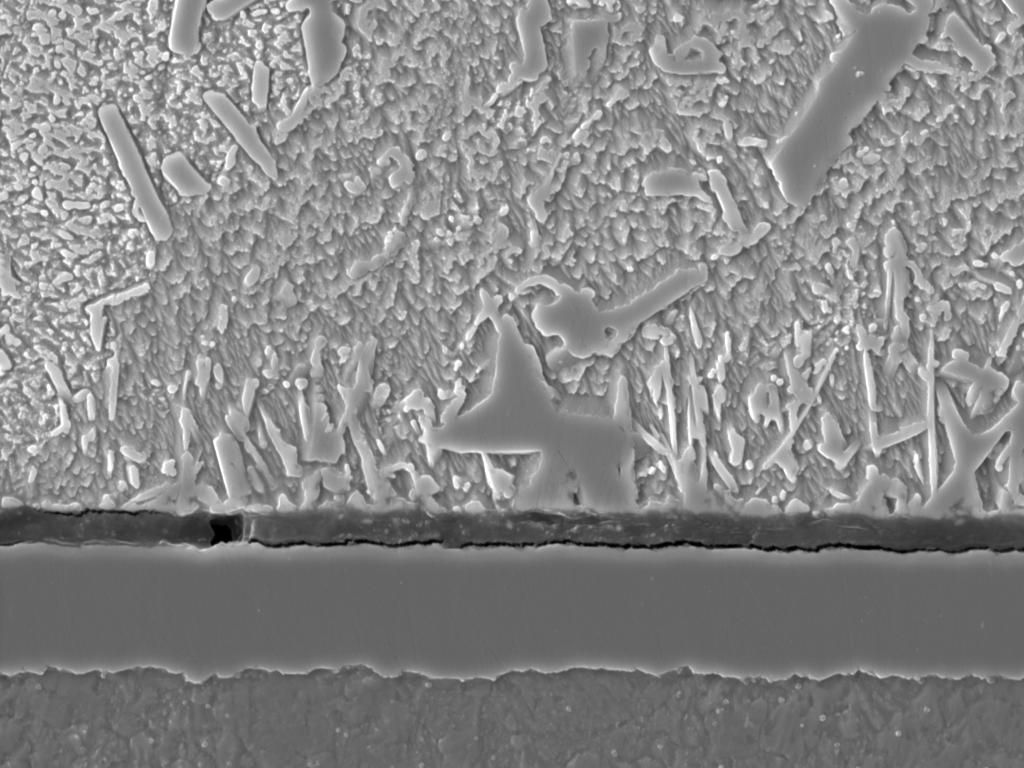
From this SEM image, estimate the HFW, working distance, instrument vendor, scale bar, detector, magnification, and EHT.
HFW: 49.9 µm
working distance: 9.99 mm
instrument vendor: Tescan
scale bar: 10000 nm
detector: SE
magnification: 5.55 K X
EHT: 20 kV